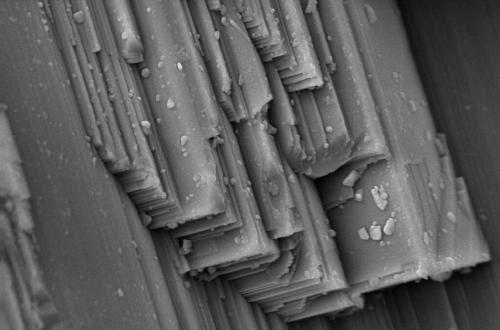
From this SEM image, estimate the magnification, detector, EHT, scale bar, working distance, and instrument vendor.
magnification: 3 K X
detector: SE1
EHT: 20 kV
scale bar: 2000 nm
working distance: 8.5 mm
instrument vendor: Zeiss